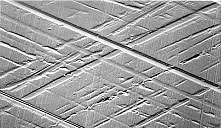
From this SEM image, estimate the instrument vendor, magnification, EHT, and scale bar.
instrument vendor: JEOL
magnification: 0.25 K X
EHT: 20 kV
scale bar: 100000 nm